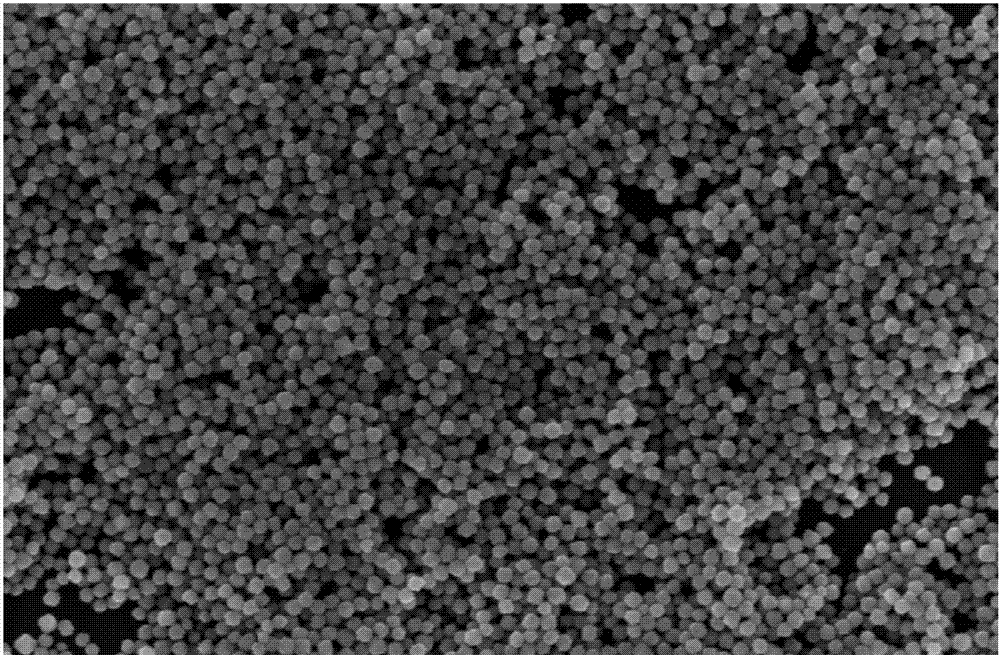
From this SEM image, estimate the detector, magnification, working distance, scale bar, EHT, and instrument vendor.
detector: TLD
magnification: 100 K X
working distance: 4.8 mm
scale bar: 1000 nm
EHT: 5 kV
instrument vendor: FEI